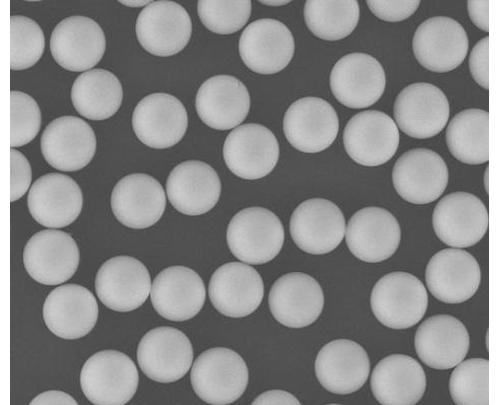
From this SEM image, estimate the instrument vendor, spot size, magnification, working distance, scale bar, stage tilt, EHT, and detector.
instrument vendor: FEI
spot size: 3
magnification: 1.2 K X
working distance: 12.3 mm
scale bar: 50000 nm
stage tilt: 0°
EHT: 20 kV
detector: LFD